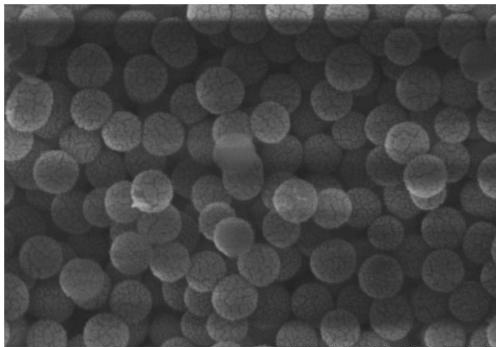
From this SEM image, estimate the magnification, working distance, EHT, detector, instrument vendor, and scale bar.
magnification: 100 K X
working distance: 9.3 mm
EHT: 10 kV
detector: SE(M)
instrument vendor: Hitachi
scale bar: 500 nm